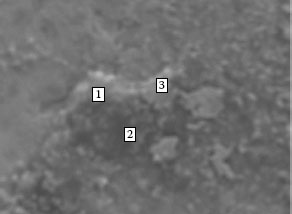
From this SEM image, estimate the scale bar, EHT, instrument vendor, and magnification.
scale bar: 10000 nm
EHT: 15 kV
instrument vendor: other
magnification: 3 K X